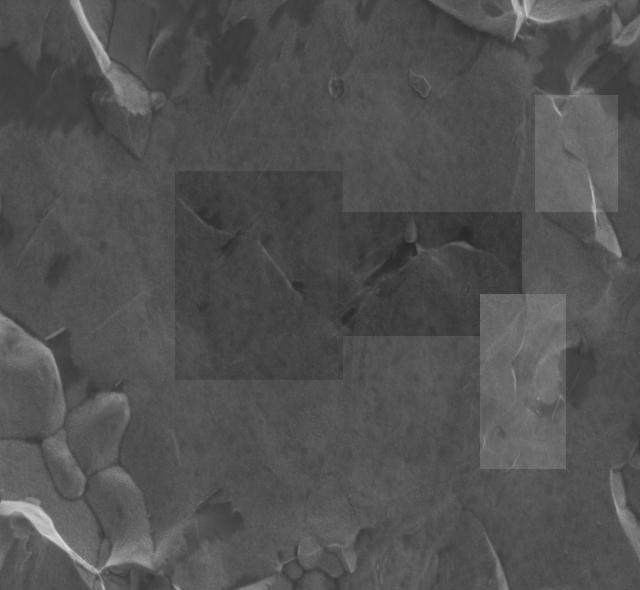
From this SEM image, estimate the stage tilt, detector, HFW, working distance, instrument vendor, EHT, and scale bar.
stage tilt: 0°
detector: ETD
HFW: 20.7 µm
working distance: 4.5 mm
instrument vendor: FEI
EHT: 2 kV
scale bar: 5000 nm